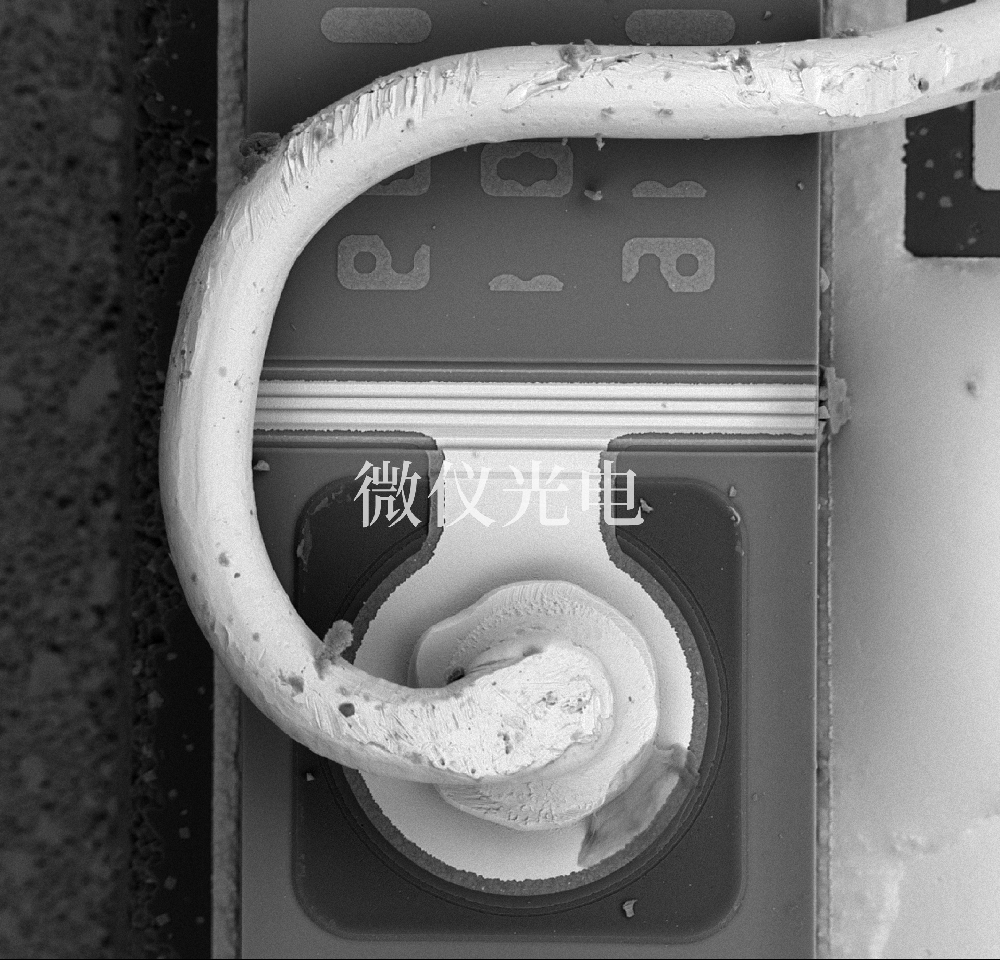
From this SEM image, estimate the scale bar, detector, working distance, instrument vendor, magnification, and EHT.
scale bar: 50000 nm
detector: BSE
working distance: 5.7 mm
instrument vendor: other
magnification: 1 K X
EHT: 15 kV